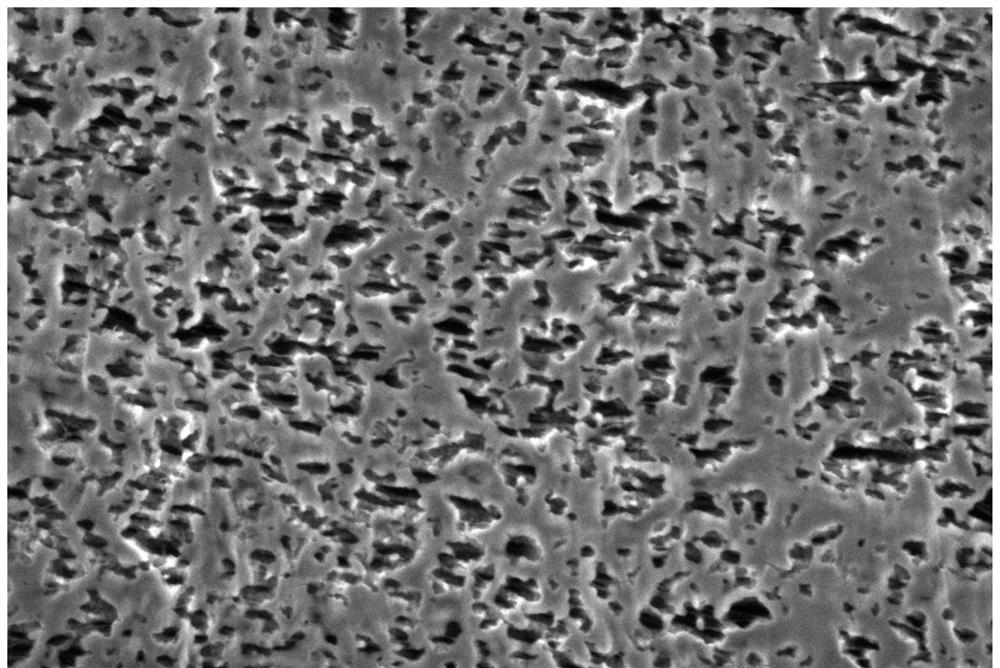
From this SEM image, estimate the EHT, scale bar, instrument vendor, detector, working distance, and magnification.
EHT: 2 kV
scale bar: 200 nm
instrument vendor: Zeiss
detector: SE2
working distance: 5.6 mm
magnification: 20 K X